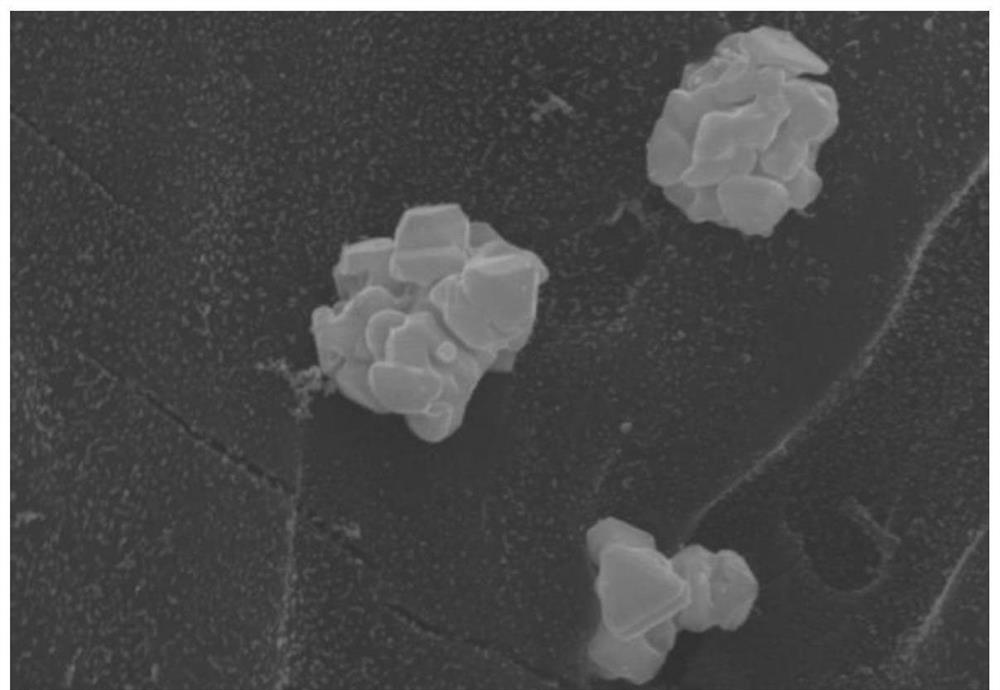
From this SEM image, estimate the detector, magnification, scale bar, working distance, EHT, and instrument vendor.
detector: SE(M)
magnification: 22 K X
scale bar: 2000 nm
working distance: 9.5 mm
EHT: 20 kV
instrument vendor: Hitachi